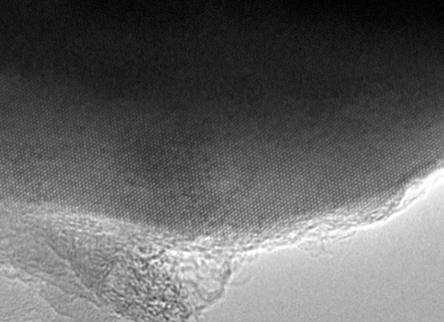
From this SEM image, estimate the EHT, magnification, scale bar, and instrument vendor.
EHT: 200 kV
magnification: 500 K X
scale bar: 10 nm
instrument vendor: JEOL